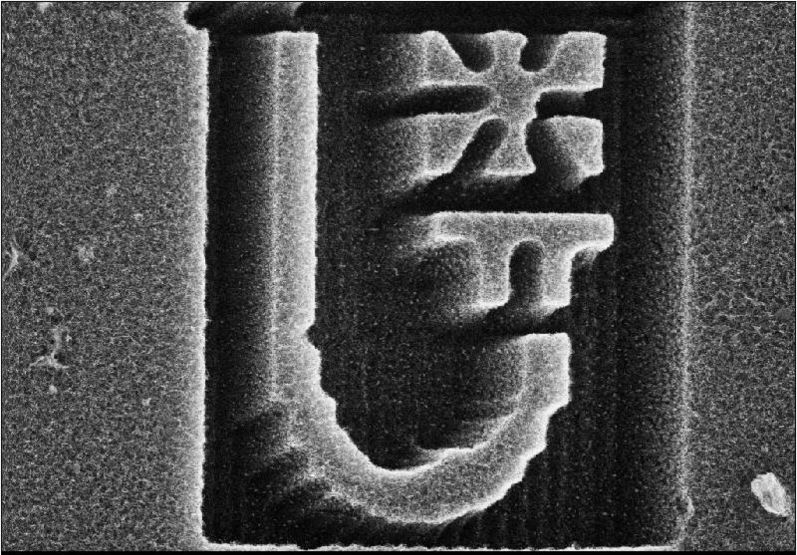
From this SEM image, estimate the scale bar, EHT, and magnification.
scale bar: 50000 nm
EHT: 25 kV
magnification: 0.8 K X